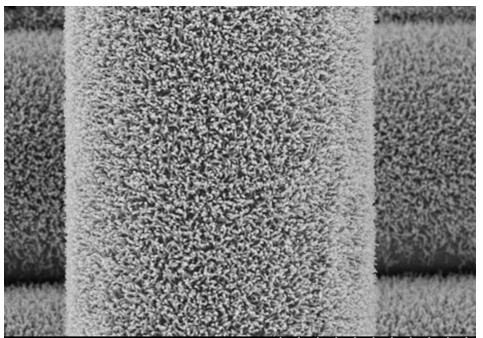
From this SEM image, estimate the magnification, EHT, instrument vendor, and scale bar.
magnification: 10 K X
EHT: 5 kV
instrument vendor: Hitachi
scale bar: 5000 nm